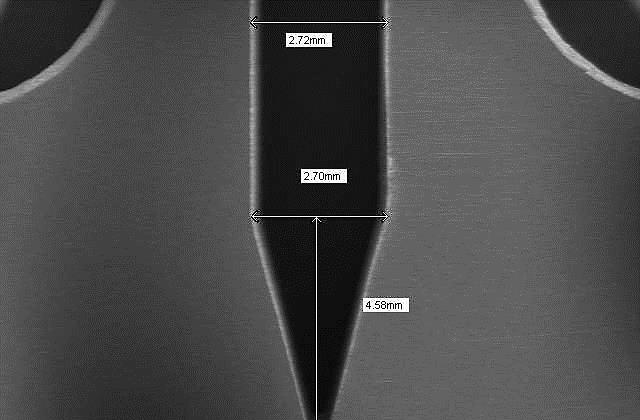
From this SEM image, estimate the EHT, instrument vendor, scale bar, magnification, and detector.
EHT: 20 kV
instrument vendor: JEOL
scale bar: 2000000 nm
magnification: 0.01 K X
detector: SEI